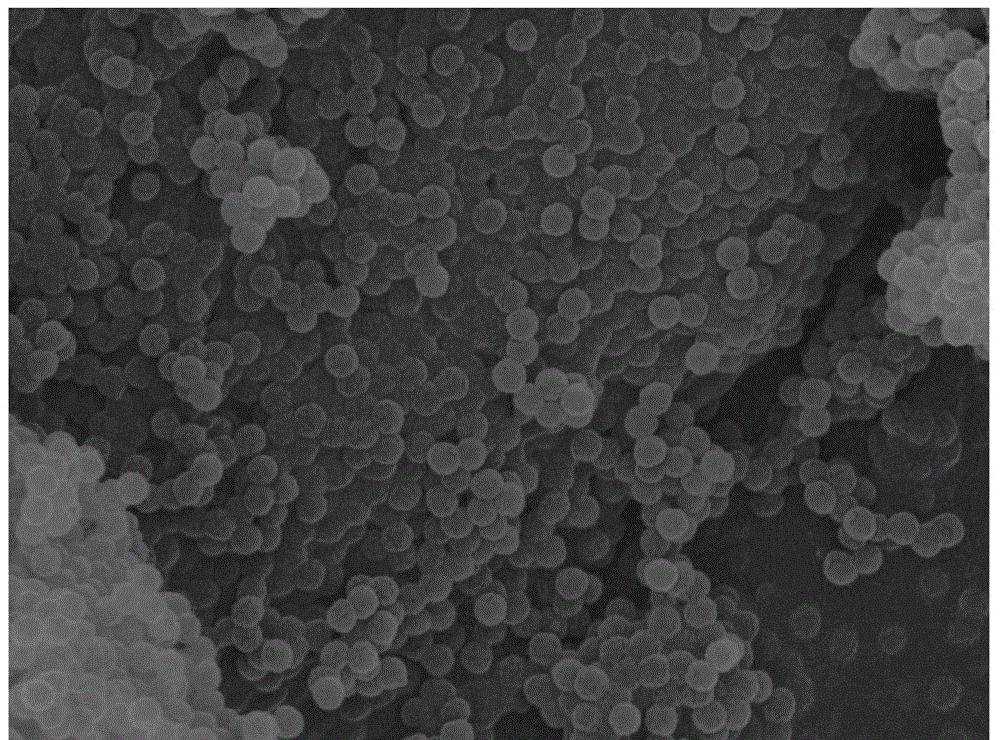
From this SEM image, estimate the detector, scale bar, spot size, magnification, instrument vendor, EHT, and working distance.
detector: TLD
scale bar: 2000 nm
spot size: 3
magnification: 10 K X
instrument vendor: FEI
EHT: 20 kV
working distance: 4.6 mm